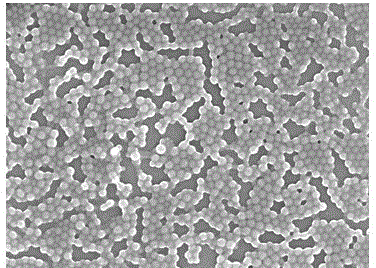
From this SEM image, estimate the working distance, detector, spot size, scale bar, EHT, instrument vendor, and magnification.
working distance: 6.4 mm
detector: TLD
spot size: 4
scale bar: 500 nm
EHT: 5 kV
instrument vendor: FEI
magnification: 40 K X